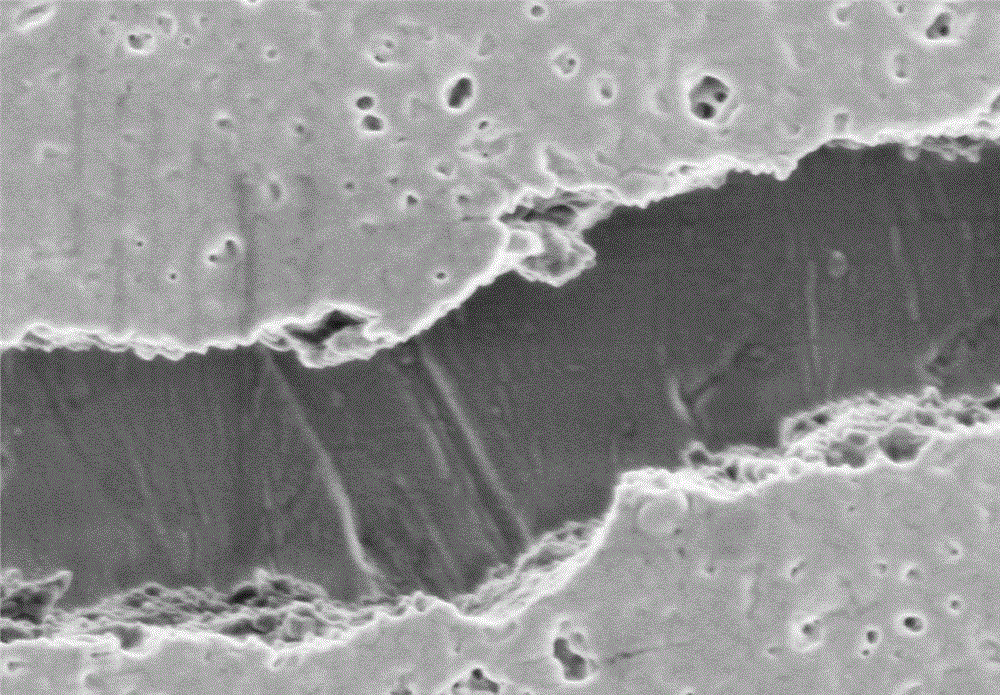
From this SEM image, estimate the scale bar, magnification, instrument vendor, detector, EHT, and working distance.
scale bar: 1000 nm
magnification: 30 K X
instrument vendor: Hitachi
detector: SE(M)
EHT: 1 kV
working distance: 9.5 mm